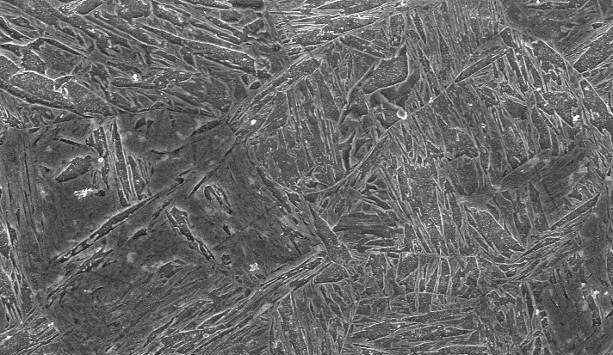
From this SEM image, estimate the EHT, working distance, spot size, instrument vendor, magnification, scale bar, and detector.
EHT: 20 kV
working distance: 11.6 mm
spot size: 4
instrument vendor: FEI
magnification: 1.5 K X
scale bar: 10000 nm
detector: SE